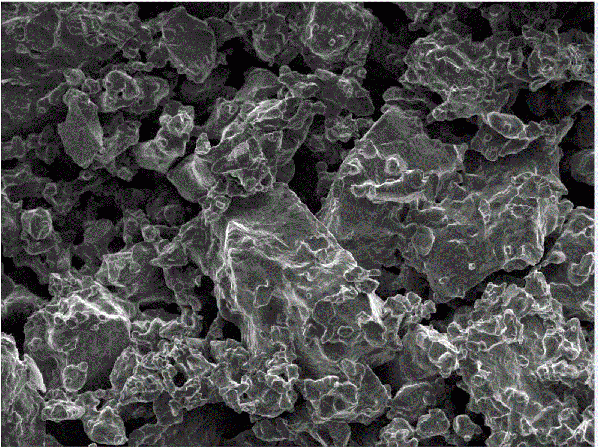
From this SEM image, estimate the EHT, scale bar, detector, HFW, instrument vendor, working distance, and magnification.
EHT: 10 kV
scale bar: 50000 nm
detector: InBeam SE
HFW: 277 µm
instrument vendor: Tescan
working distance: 4.98 mm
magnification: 1 K X